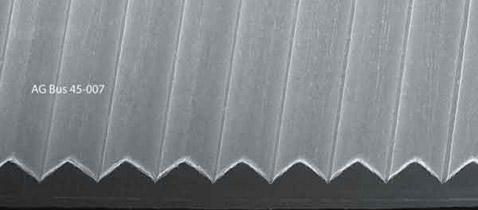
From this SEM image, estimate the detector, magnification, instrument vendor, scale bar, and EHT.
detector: SE1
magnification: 0.1 K X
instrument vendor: Zeiss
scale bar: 100000 nm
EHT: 20 kV